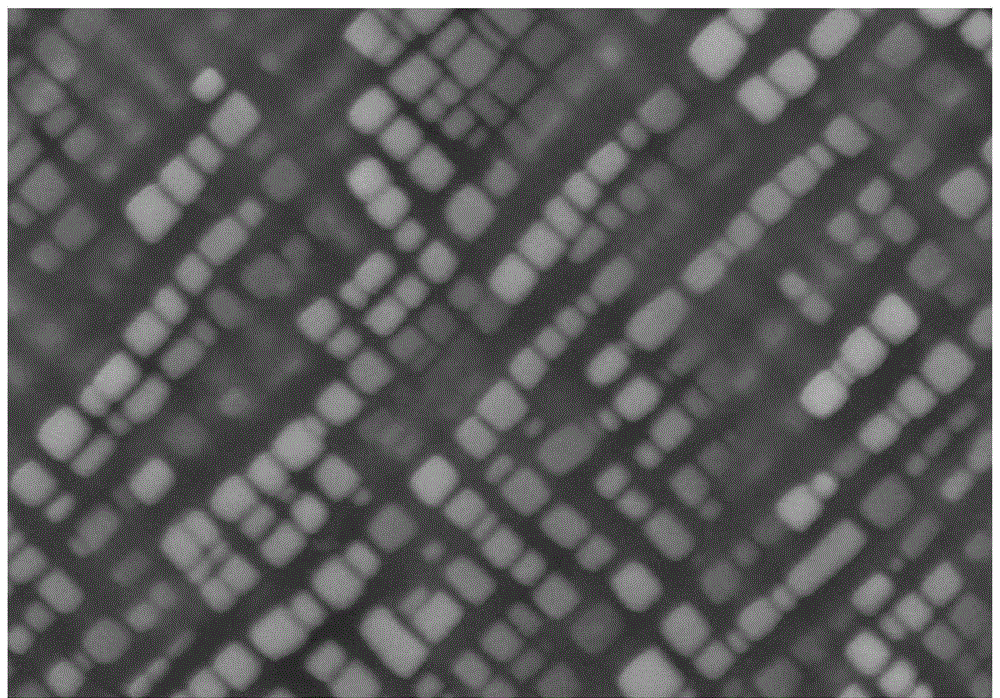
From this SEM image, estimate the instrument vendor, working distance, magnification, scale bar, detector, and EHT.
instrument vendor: Zeiss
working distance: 7.7 mm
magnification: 50 K X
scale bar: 100 nm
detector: SE2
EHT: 10 kV